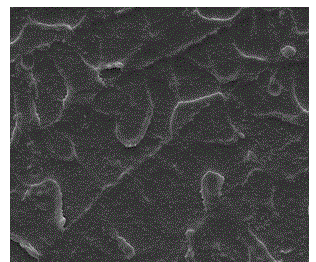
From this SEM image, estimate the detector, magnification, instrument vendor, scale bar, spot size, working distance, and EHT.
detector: ETD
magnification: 15 K X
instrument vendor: FEI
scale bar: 4000 nm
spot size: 3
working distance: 5.8 mm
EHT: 10 kV